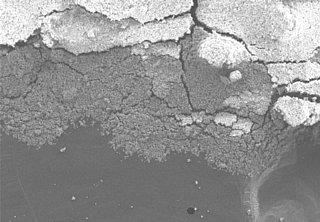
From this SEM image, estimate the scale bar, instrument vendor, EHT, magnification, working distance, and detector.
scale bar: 50000 nm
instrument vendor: Hitachi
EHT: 5 kV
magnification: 0.2 K X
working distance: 6.7 mm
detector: S